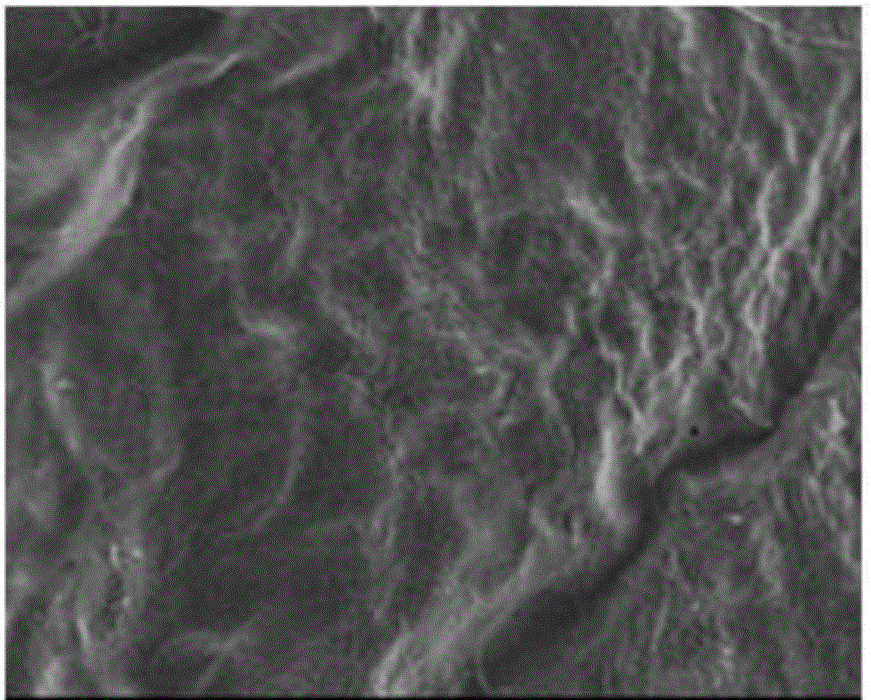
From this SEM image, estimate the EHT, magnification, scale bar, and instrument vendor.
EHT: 15 kV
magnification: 0.1 K X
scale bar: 100000 nm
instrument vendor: Zeiss